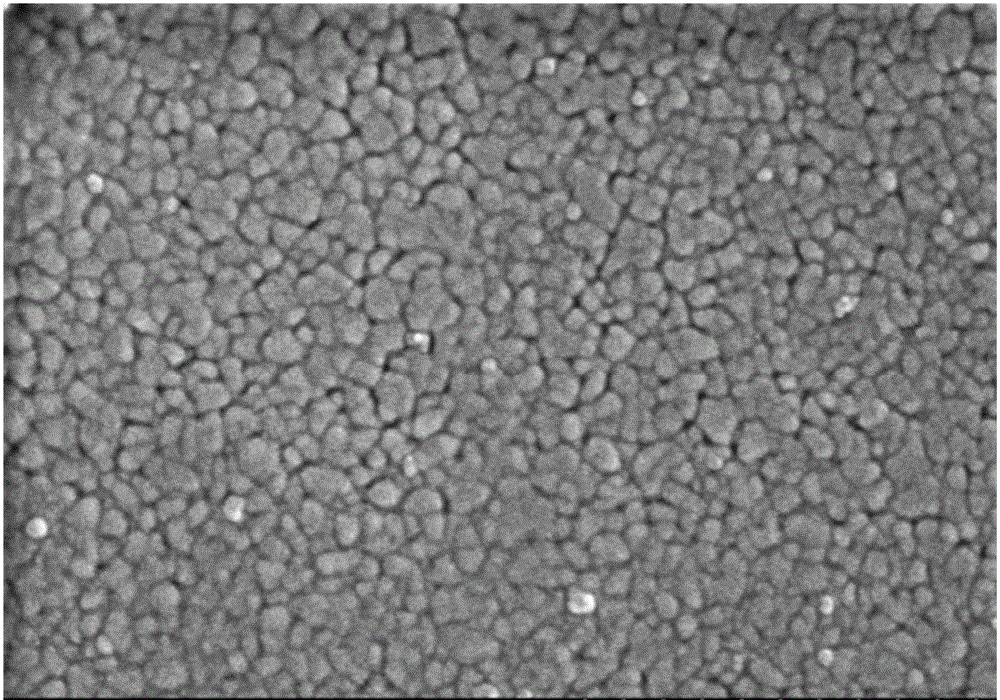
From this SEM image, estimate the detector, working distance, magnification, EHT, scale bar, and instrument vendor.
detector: SE(M)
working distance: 7.1 mm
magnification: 50 K X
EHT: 3 kV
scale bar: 1000 nm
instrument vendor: Hitachi